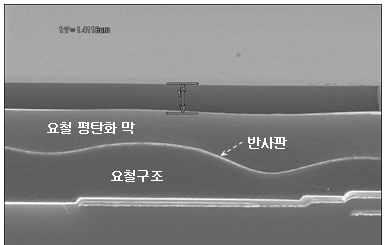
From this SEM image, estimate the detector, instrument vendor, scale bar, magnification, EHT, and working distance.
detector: SE(U)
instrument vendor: Hitachi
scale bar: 5000 nm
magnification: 7 K X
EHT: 15 kV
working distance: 16.4 mm